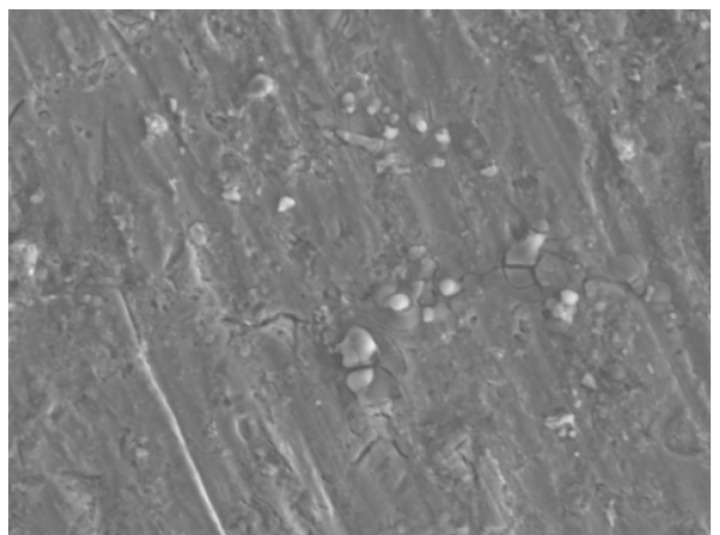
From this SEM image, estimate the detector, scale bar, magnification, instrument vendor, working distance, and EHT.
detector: SE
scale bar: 20000 nm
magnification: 1.34 K X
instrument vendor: Tescan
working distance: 14.39 mm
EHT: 18 kV